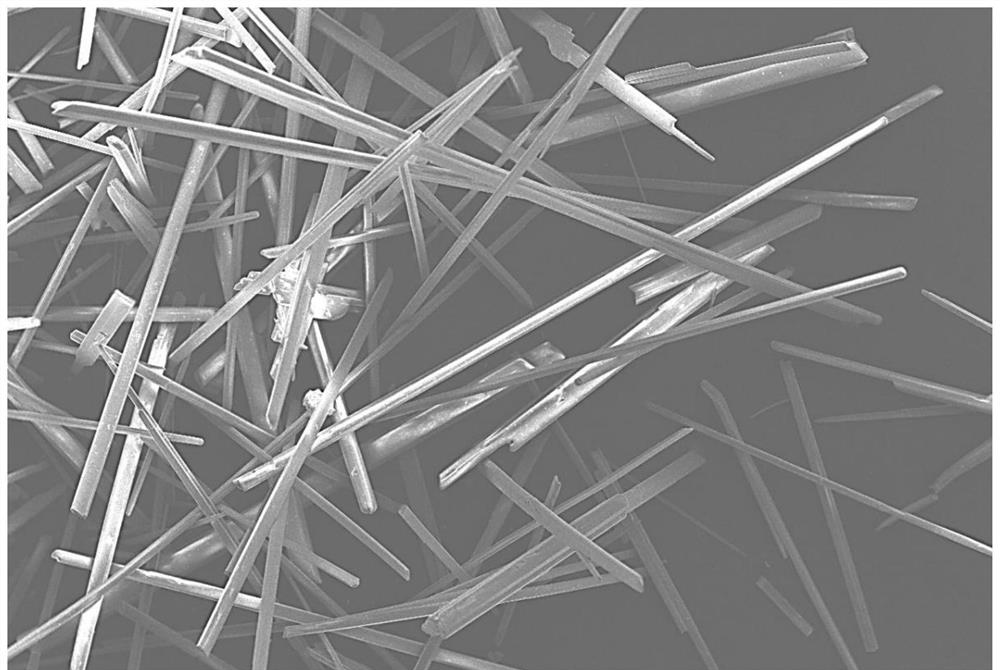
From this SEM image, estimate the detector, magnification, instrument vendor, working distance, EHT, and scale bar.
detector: LED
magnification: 0.05 K X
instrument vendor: JEOL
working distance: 14.2 mm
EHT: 10 kV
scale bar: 100000 nm